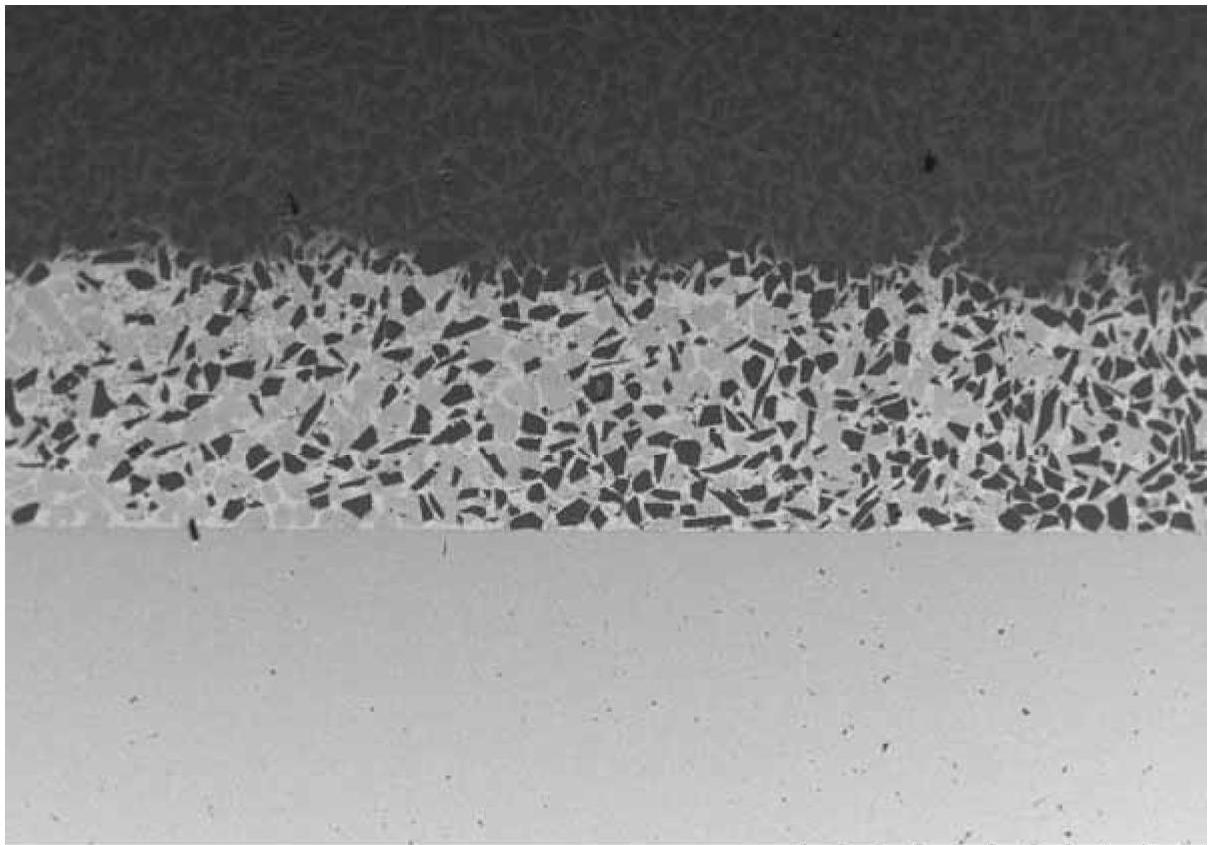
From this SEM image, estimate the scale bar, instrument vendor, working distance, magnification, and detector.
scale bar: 1000000 nm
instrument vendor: Hitachi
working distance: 10.3 mm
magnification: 0.04 K X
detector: BSE3D